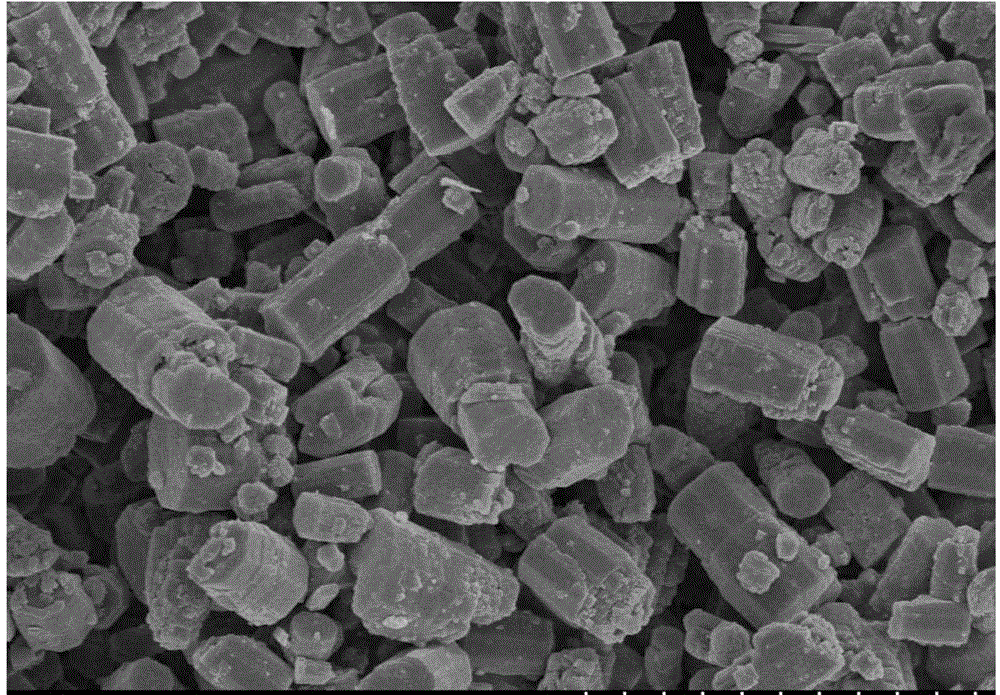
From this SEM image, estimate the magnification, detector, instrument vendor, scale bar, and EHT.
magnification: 5 K X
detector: SE(M)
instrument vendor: Hitachi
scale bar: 10000 nm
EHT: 5 kV